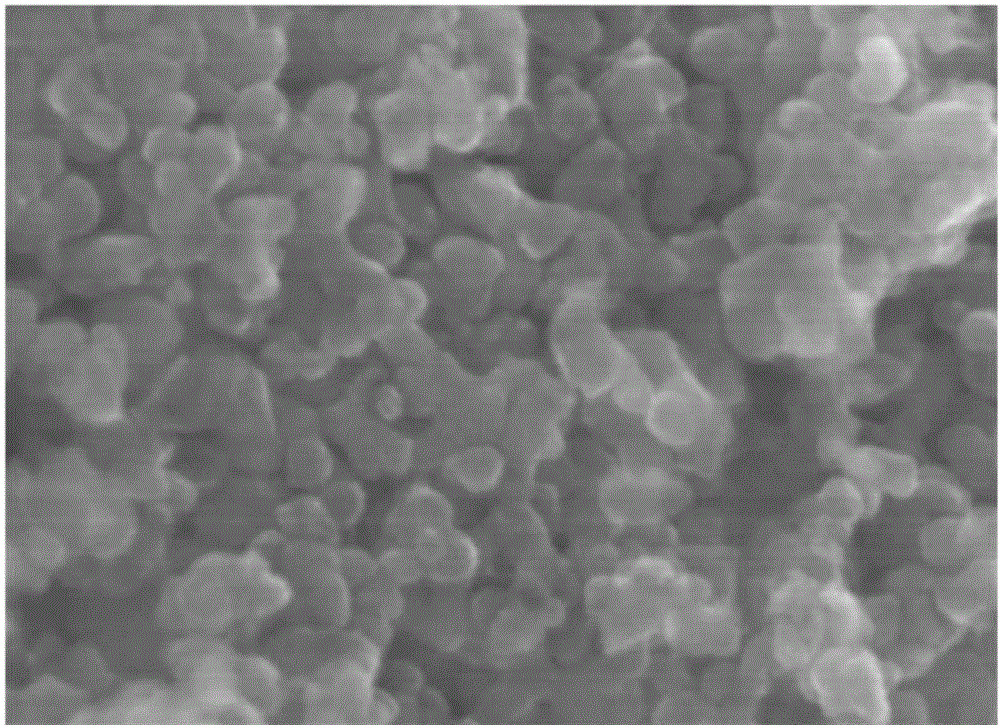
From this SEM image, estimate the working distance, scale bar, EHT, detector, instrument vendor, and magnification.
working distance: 4.1 mm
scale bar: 200 nm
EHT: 15 kV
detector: SE(UL)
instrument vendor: Hitachi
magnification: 200 K X